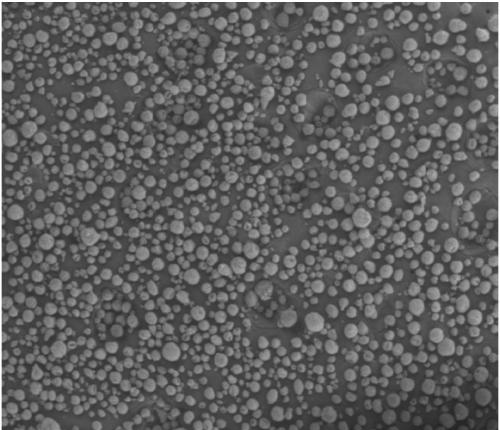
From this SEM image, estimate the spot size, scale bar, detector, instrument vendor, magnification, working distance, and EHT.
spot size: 3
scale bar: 500000 nm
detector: LFD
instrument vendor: FEI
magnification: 0.1 K X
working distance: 9.1 mm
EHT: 20 kV